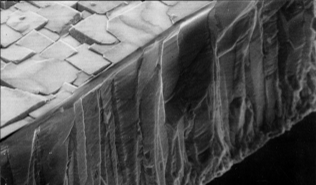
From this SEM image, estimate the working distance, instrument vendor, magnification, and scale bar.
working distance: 14 mm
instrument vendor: JEOL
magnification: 8.5 K X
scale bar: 1000 nm